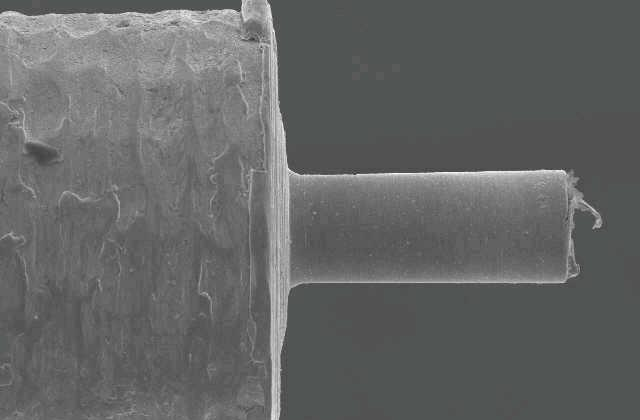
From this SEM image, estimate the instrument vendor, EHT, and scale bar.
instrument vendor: JEOL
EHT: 20 kV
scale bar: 100000 nm